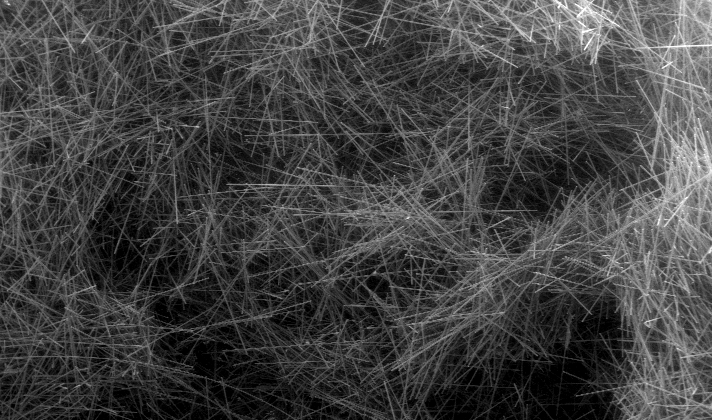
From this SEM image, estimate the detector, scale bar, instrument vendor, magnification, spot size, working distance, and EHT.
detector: GSE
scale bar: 1000000 nm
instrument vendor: FEI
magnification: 0.03 K X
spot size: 4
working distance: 20.5 mm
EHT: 20 kV